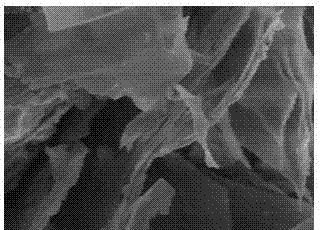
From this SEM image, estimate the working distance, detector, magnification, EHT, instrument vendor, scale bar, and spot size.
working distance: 5.4 mm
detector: TLD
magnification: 10 K X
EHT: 5 kV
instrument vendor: FEI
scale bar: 2000 nm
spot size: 4